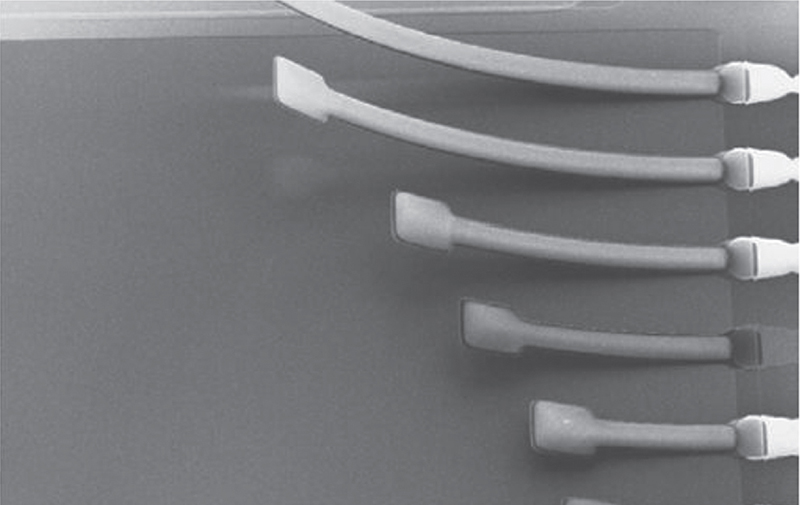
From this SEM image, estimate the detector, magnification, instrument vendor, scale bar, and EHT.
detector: SE1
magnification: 0.117 K X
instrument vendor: Zeiss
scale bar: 300000 nm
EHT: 10 kV